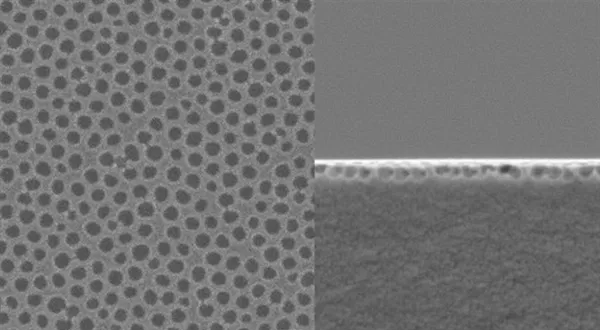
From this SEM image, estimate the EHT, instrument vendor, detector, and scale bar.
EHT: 2 kV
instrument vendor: JEOL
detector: SEI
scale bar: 1000 nm